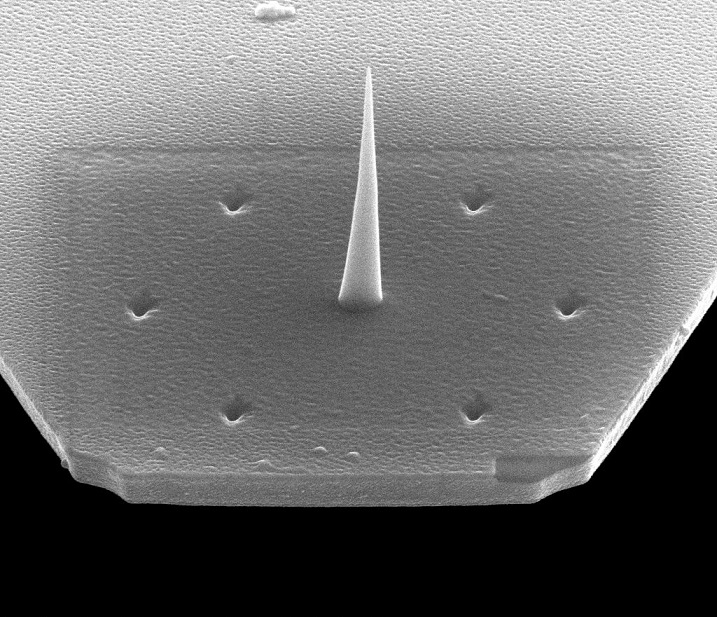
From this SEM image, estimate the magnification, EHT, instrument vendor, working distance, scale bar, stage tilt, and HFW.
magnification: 19.994 K X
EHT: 20 kV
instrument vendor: FEI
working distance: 4.1 mm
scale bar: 2000 nm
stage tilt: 52°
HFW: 7.46 µm